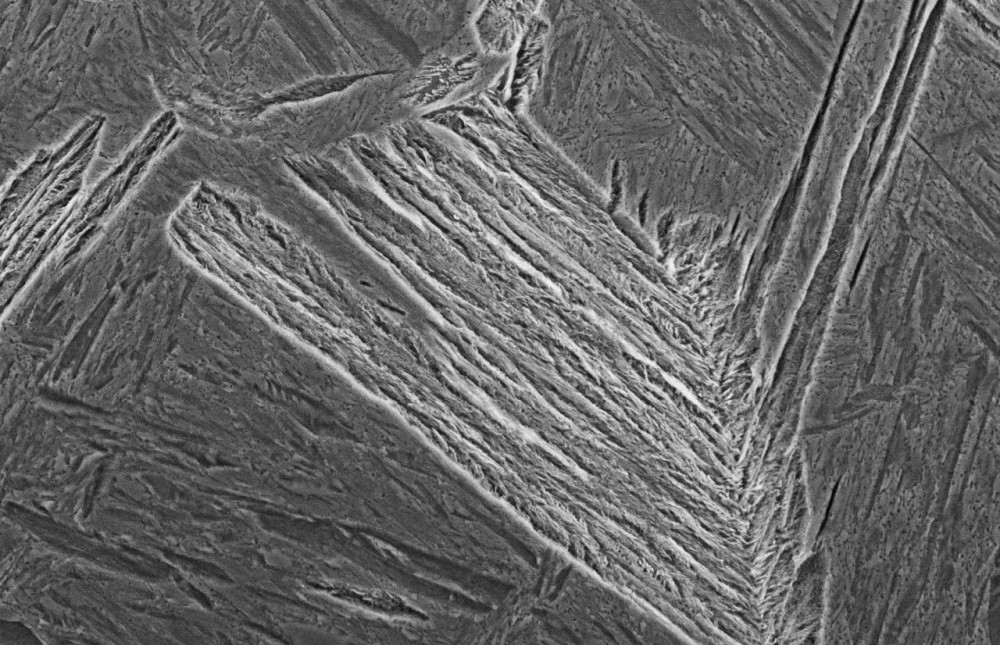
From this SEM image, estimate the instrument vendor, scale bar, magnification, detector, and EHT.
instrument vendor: JEOL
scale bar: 10000 nm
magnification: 2 K X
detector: SEI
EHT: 15 kV